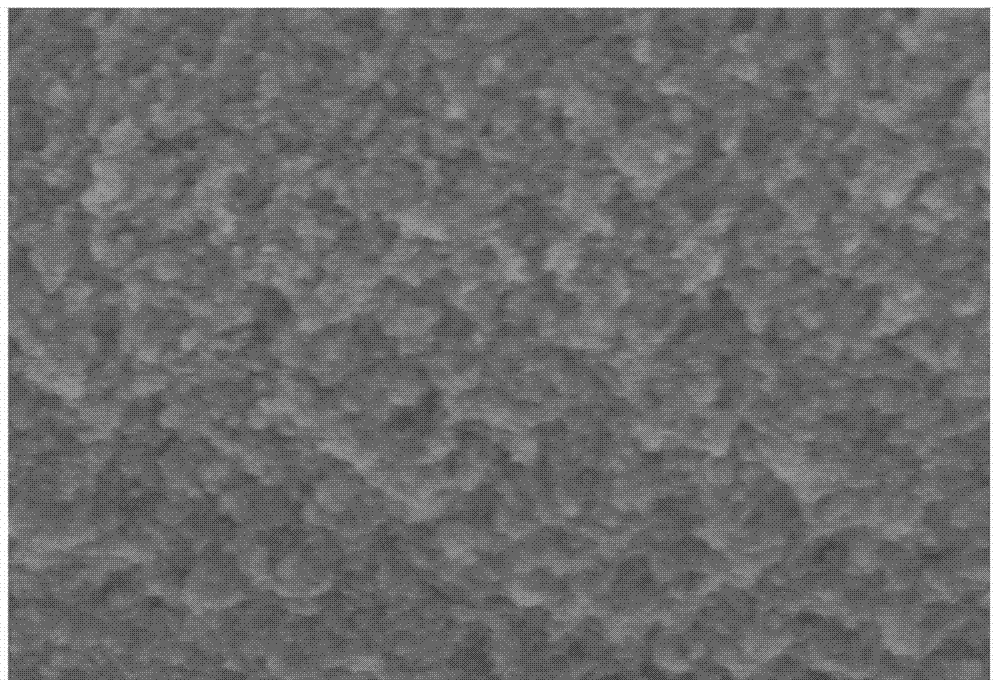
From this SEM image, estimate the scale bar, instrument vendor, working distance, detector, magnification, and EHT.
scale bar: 1000 nm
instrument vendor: Hitachi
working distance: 6.3 mm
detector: SE(U)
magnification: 50 K X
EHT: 2 kV